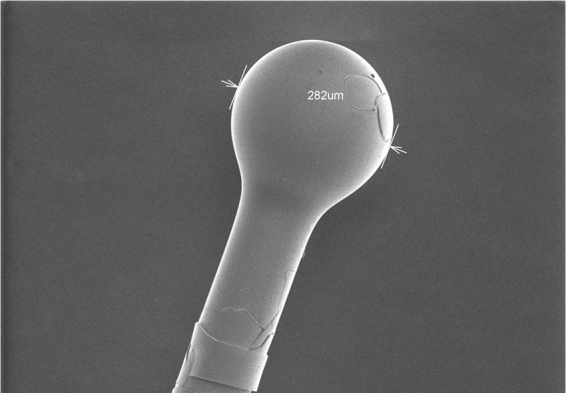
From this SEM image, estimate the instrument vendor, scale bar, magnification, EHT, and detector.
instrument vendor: Hitachi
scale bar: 400000 nm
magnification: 0.13 K X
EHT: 2 kV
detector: SE